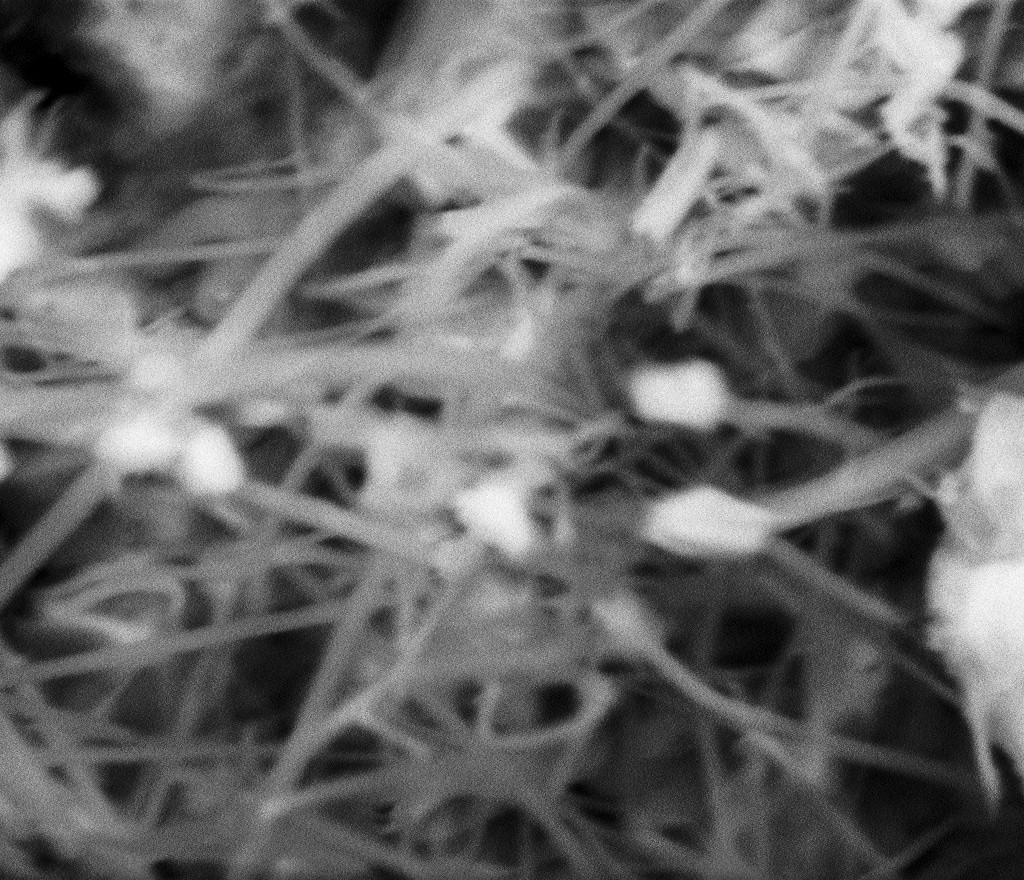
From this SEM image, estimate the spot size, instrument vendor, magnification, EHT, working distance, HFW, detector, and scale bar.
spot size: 5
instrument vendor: FEI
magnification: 35 K X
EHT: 10 kV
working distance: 4.3 mm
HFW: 4.26 µm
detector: BSED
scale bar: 1000 nm